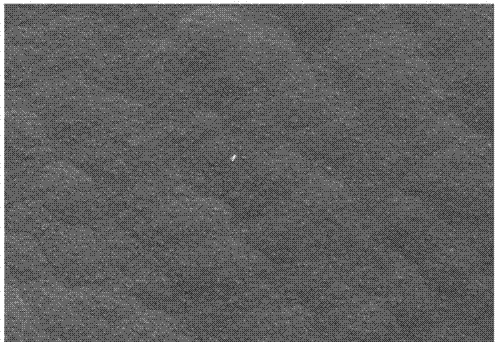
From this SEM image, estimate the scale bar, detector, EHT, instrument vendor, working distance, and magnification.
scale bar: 1000 nm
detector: SE2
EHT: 5 kV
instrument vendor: Zeiss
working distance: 16.3 mm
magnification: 3 K X